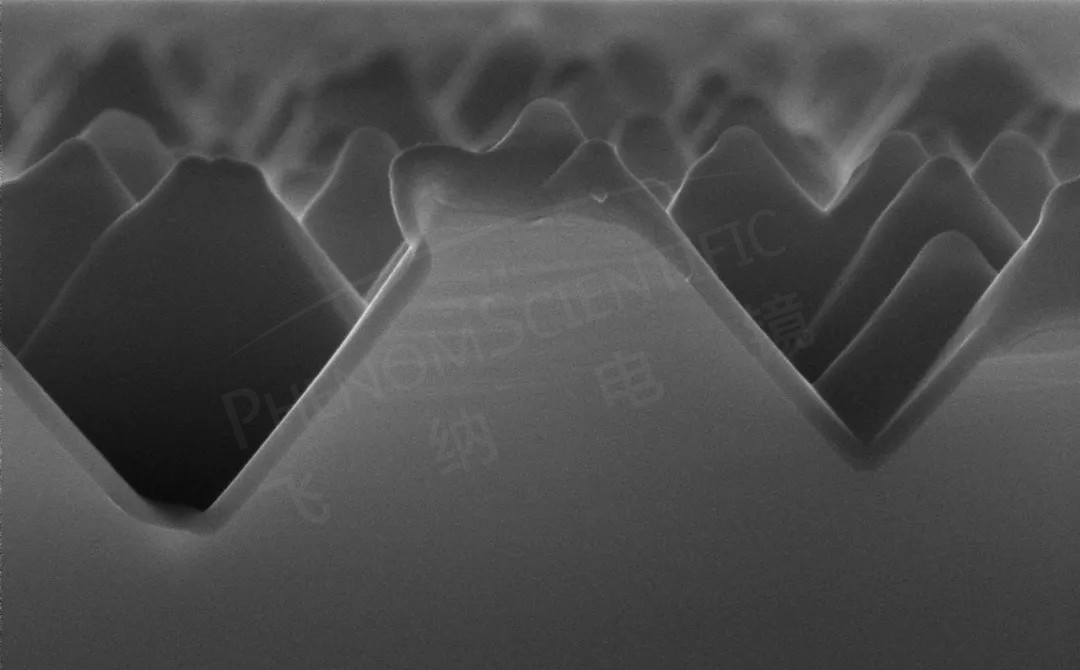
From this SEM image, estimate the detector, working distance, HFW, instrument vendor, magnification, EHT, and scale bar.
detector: SED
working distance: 7.747 mm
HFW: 4.17 µm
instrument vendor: other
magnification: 30 K X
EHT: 15 kV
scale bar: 1000 nm